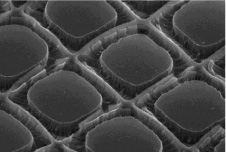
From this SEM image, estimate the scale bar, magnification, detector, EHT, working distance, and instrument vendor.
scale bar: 100000 nm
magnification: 0.4 K X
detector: SE(U)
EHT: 20 kV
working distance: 14.2 mm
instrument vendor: Hitachi